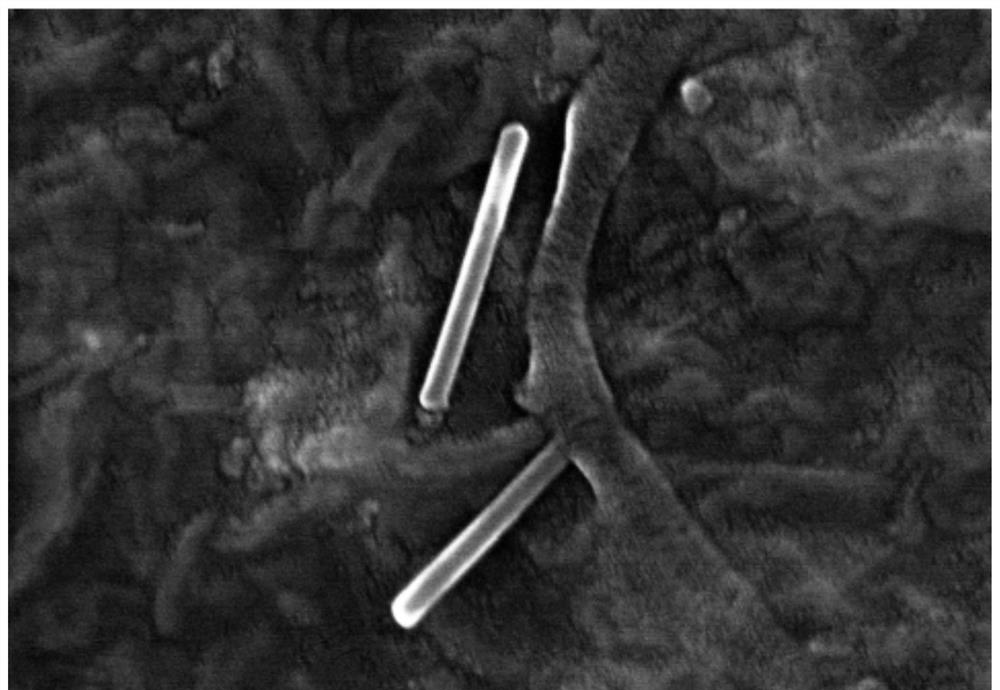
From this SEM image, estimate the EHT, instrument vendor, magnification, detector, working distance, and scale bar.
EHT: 10 kV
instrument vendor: Hitachi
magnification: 70 K X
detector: SE(UL)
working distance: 8.8 mm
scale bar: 500 nm